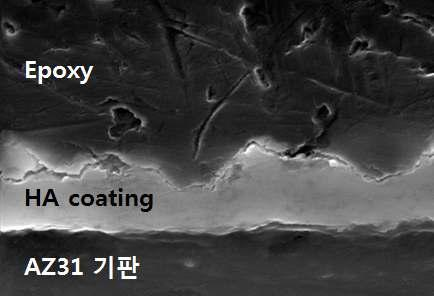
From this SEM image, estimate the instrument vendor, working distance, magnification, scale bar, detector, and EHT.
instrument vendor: Zeiss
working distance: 10 mm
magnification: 10 K X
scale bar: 3000 nm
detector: SE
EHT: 20 kV